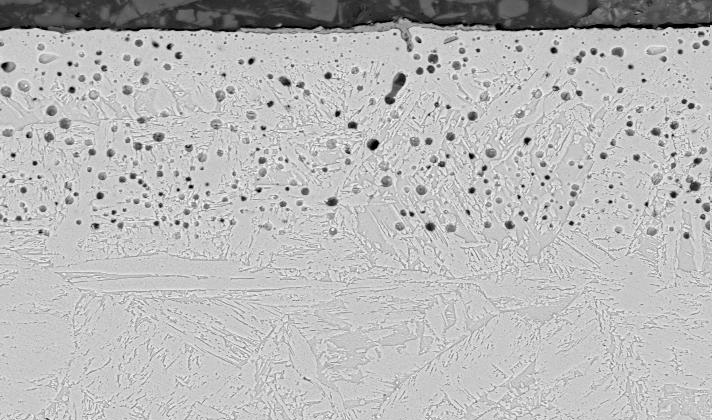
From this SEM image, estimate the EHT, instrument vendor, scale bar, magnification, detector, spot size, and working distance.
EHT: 20 kV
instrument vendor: FEI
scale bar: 20000 nm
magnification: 1.5 K X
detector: BSE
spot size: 5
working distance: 10.6 mm